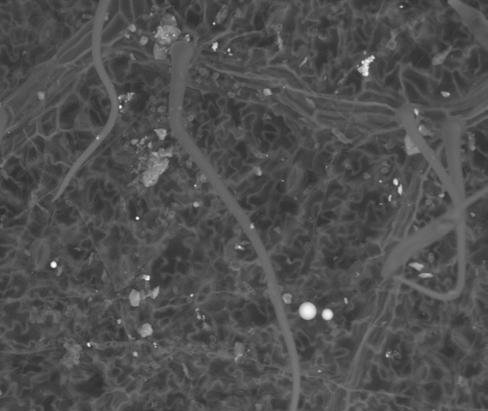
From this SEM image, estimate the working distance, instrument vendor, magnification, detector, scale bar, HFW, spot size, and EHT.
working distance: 10.1 mm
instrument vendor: FEI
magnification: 0.8 K X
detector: SSD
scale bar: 100000 nm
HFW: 340 µm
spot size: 5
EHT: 25 kV